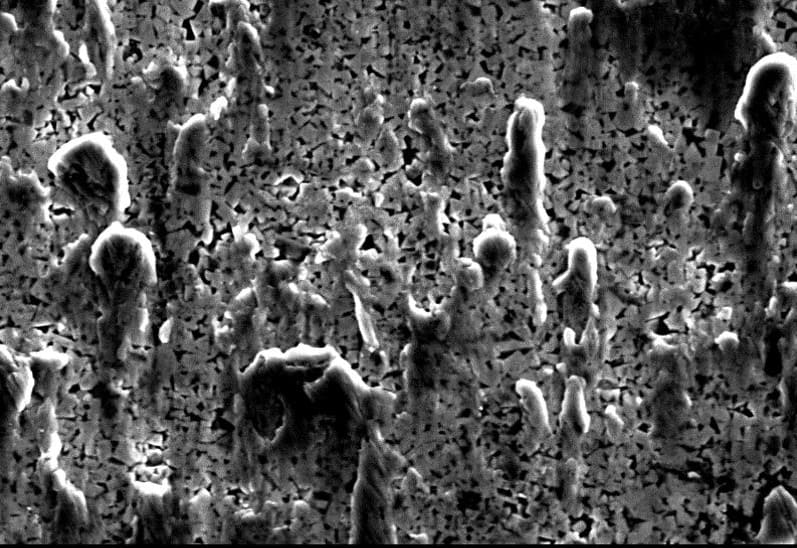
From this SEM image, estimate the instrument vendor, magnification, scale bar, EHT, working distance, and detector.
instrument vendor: JEOL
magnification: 2 K X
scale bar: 10000 nm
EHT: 20 kV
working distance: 10 mm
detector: SEI